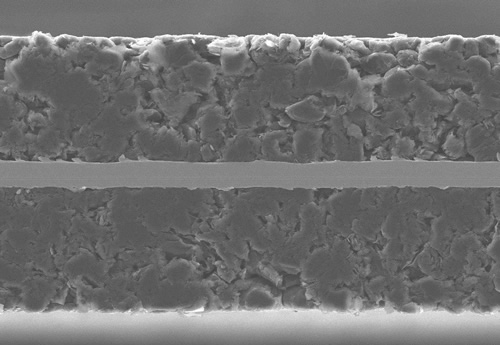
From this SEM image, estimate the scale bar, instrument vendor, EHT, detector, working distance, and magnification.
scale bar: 100000 nm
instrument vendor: Hitachi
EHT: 15 kV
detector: SE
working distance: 8.5 mm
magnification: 0.5 K X